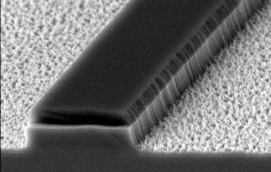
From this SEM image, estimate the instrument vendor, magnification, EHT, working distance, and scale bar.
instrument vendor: JEOL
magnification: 35 K X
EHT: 5 kV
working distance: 19 mm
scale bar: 1000 nm